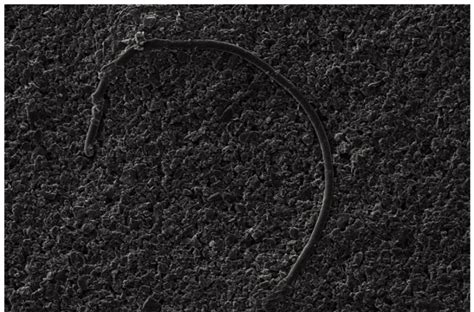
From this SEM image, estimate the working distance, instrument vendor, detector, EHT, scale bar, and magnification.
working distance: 9 mm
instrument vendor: Zeiss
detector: SE1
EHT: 10 kV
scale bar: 100000 nm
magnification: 0.2 K X